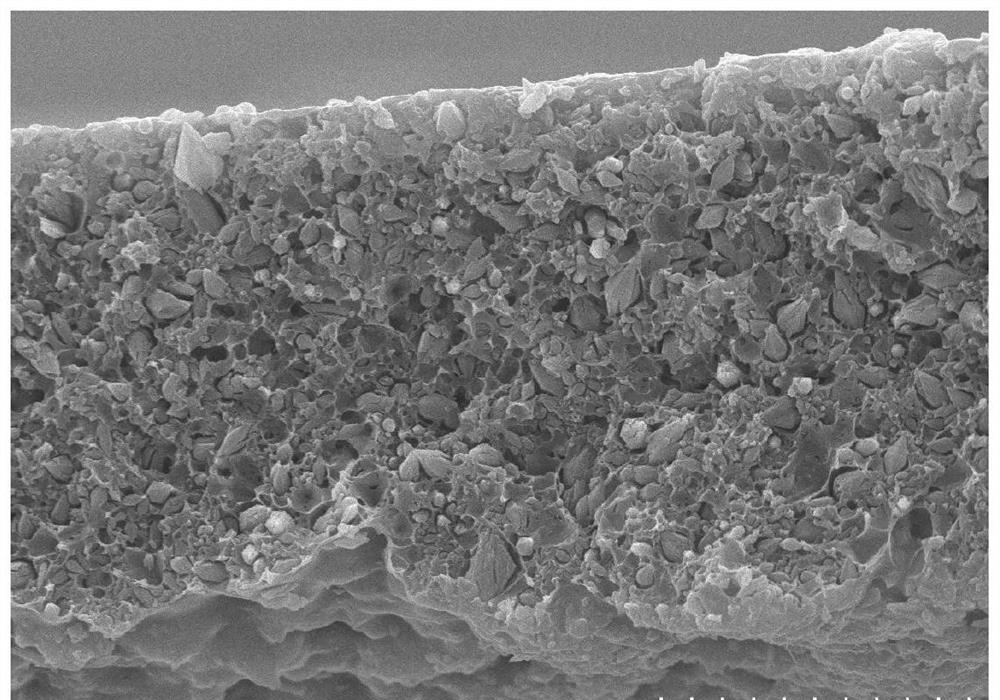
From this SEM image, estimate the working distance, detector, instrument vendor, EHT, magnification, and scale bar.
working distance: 10.1 mm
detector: SE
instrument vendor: Hitachi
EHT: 20 kV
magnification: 2 K X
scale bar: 20000 nm